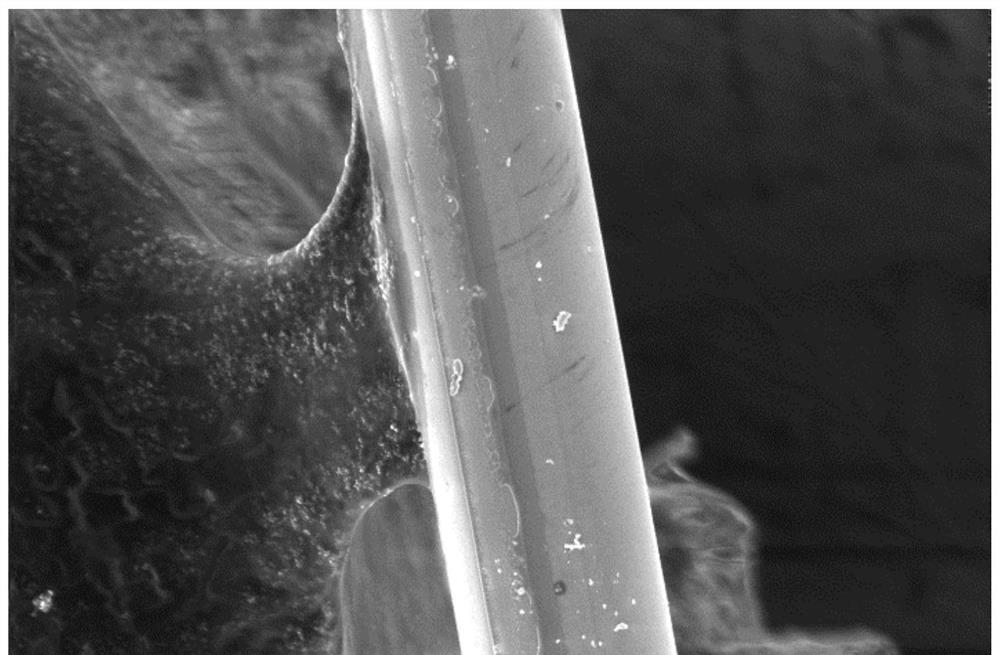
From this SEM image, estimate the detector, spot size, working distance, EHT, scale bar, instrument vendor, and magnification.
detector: TLD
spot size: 4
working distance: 6.1 mm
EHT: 5 kV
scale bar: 10000 nm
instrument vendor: FEI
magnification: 5 K X